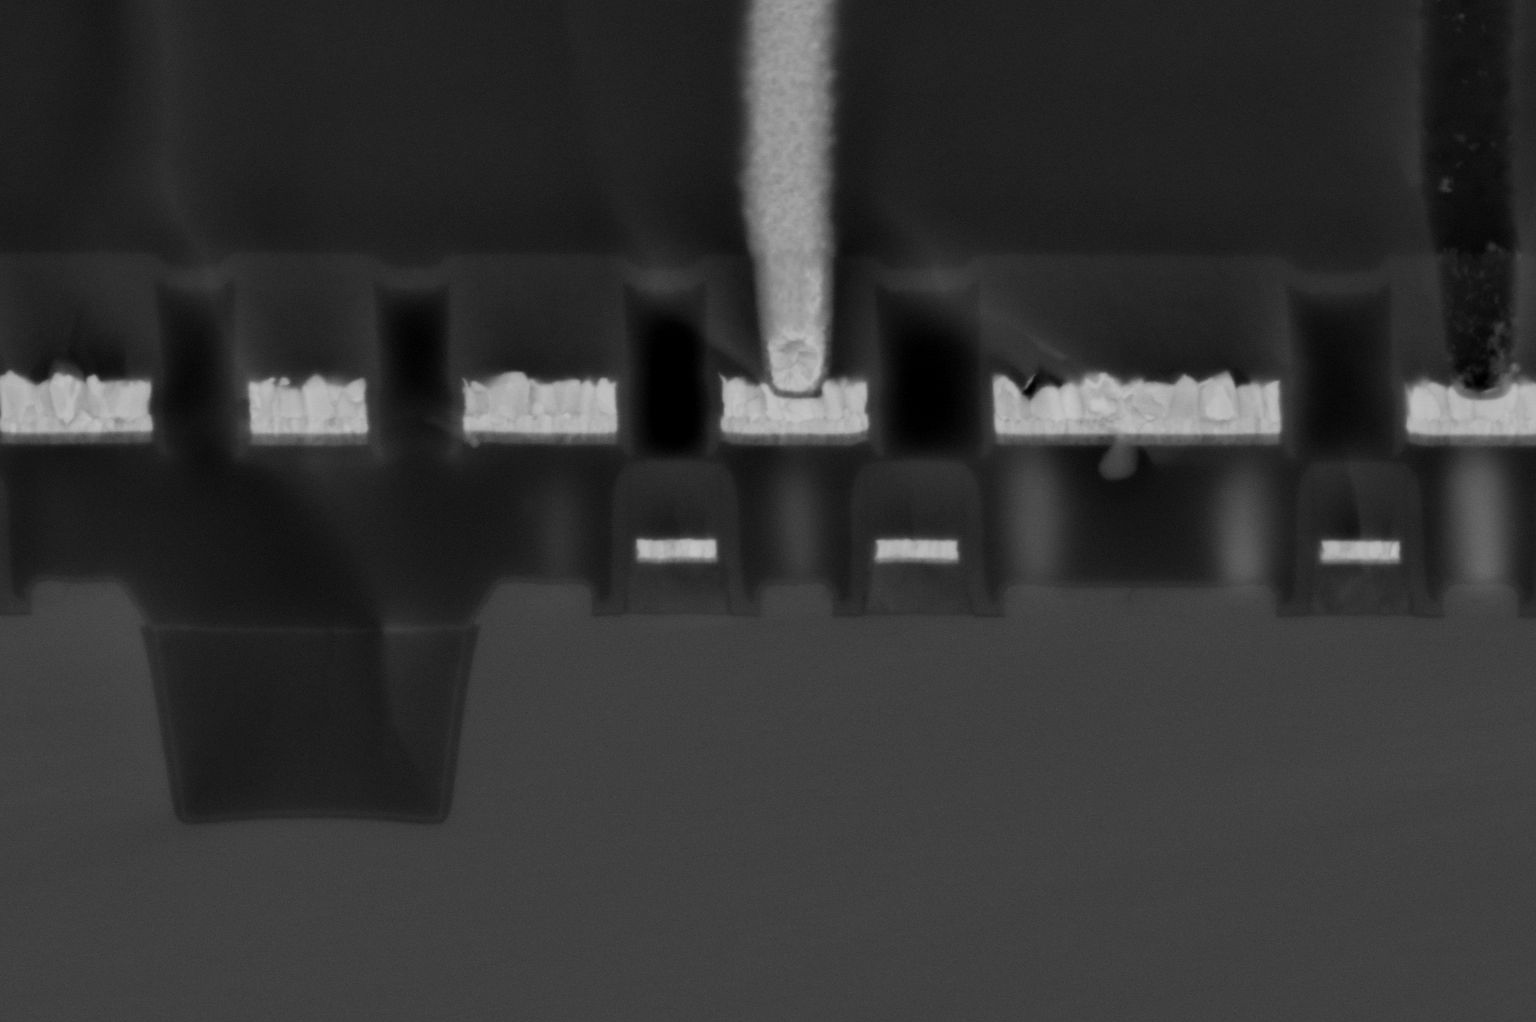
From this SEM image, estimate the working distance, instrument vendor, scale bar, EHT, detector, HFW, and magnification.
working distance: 1.2 mm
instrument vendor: FEI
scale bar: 500 nm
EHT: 3 kV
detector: MD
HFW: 1.95 µm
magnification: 65 K X